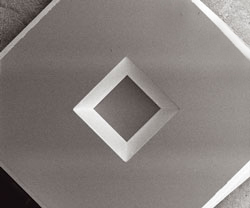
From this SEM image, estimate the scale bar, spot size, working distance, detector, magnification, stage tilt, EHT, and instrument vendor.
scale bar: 500000 nm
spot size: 3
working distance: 5.1 mm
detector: SED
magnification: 0.117 K X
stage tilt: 0°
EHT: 2 kV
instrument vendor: FEI